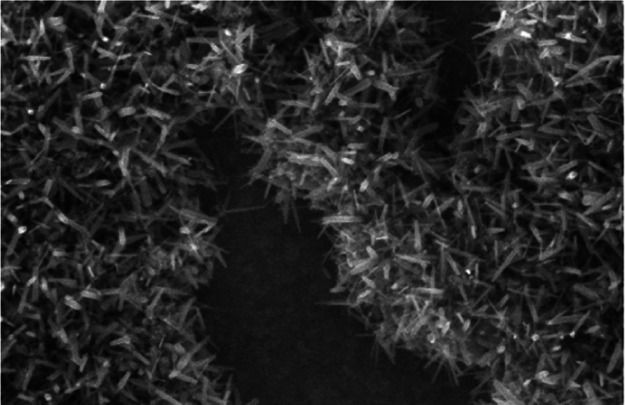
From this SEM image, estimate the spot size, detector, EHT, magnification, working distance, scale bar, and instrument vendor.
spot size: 3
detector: TLD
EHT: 5 kV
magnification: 50 K X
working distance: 2.8 mm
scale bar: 500 nm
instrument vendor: FEI